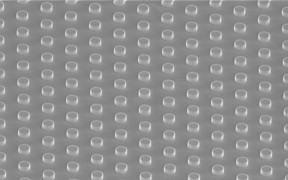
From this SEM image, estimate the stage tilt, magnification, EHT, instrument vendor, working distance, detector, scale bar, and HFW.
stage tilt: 52°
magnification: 10 K X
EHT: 2 kV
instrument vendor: FEI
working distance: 3.8 mm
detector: TLD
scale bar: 5000 nm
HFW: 20.7 µm